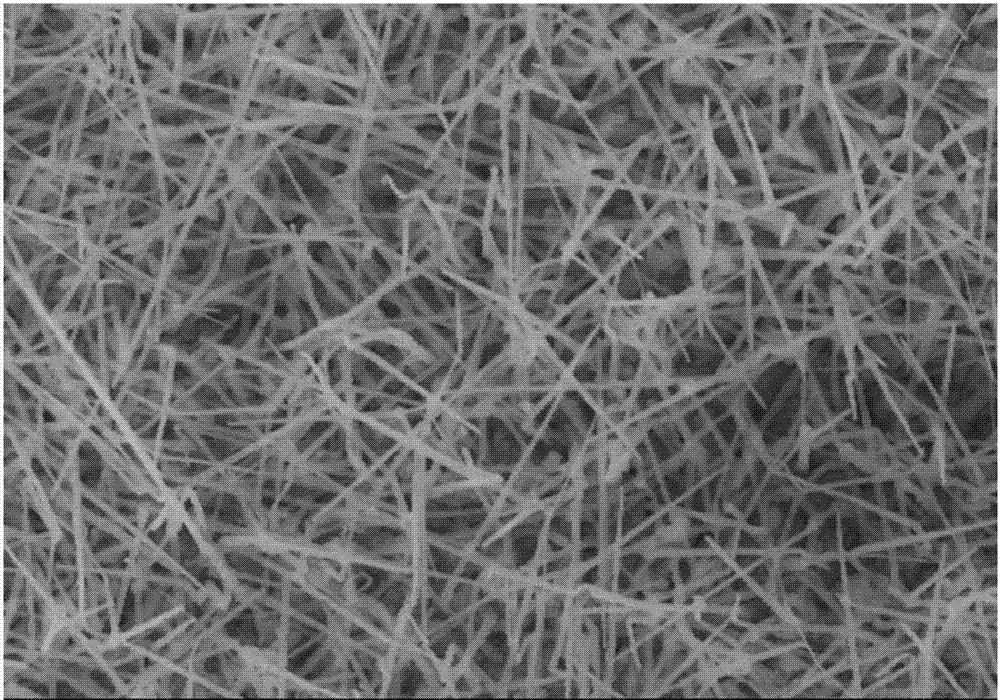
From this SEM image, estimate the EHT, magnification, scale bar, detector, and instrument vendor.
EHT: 10 kV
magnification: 20 K X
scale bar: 2000 nm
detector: SE(M)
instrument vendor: Hitachi